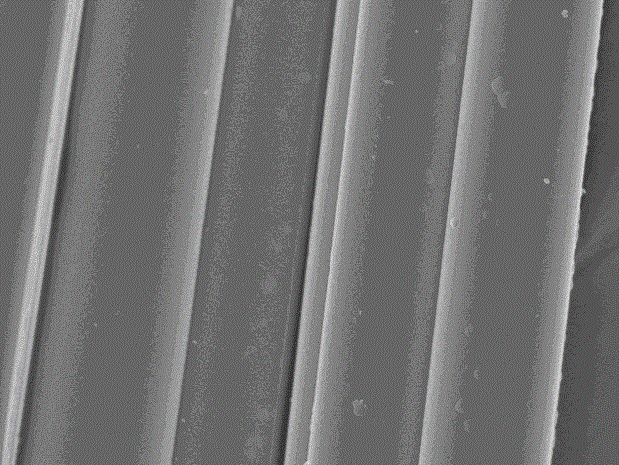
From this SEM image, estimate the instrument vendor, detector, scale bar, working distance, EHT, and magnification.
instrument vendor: JEOL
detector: SEI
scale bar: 10000 nm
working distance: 11.2 mm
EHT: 15 kV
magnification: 2 K X